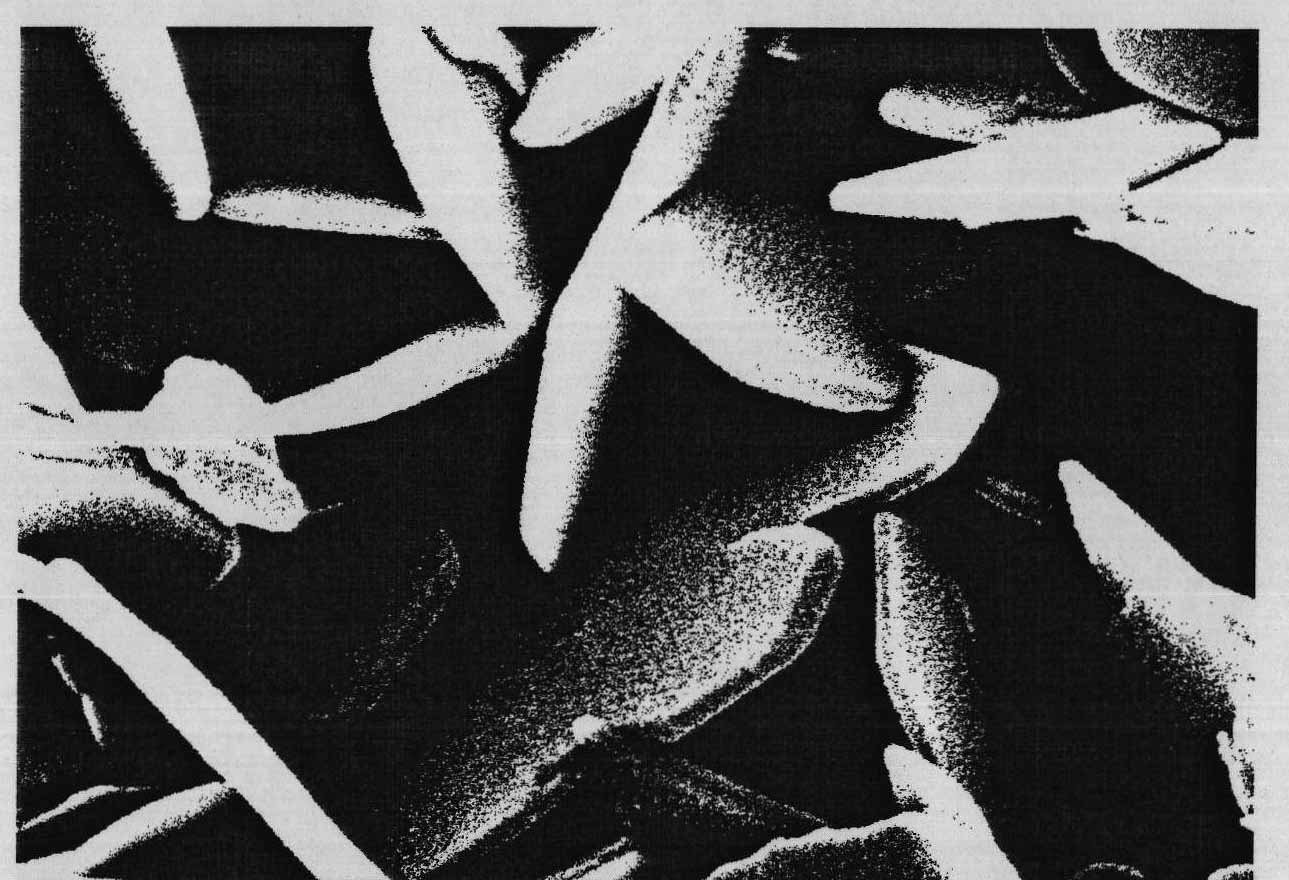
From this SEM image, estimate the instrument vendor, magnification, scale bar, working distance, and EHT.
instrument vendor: Hitachi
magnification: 100 K X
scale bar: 500 nm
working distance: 11 mm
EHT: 20 kV